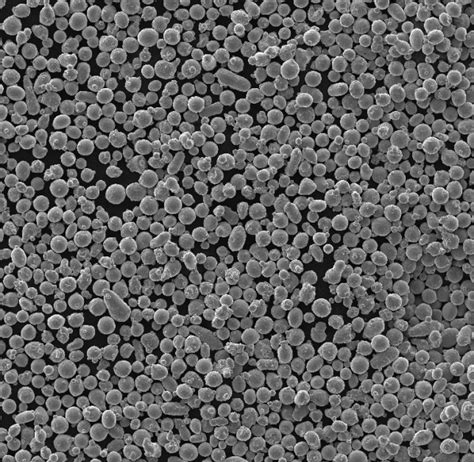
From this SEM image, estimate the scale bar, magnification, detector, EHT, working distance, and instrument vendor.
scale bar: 200000 nm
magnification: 0.2 K X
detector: SE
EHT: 20 kV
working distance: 12.99 mm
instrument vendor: Tescan